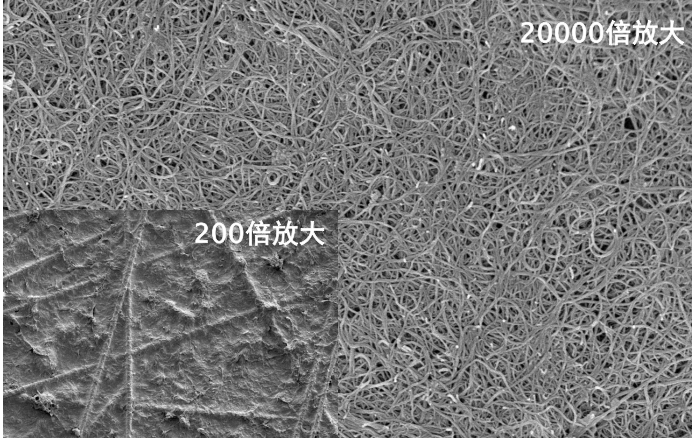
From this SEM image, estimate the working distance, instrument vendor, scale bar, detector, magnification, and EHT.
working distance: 3.8 mm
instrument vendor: Hitachi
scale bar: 2000 nm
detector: SE(UL)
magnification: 20 K X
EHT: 3 kV